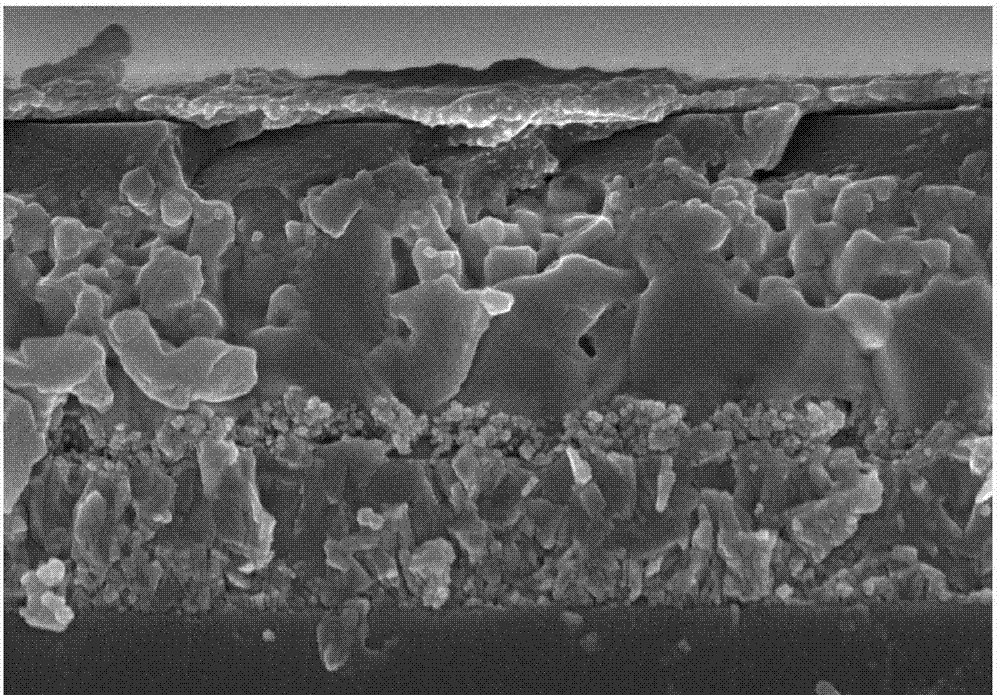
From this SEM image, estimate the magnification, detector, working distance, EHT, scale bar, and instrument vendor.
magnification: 30 K X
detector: SE(M)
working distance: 6.4 mm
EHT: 5 kV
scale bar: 1000 nm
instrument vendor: Hitachi